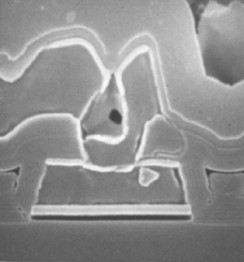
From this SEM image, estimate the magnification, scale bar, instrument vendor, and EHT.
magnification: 24.9 K X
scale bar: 1200 nm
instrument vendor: JEOL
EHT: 20 kV